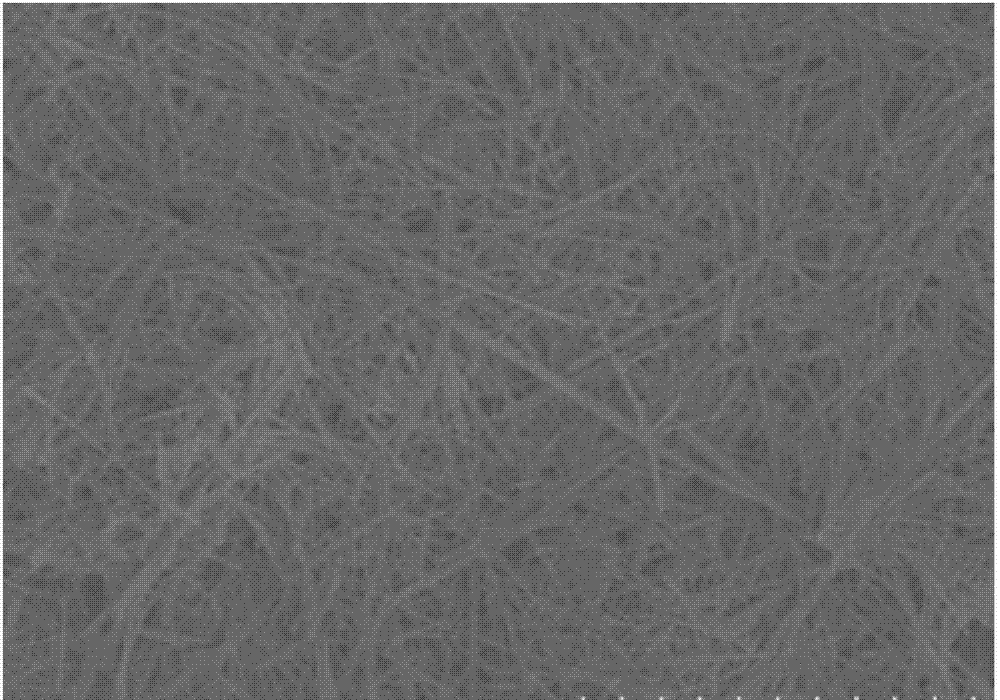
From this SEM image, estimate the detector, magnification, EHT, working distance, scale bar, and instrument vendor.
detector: SE(M)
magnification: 5 K X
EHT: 3 kV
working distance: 8.6 mm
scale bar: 10000 nm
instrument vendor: Hitachi